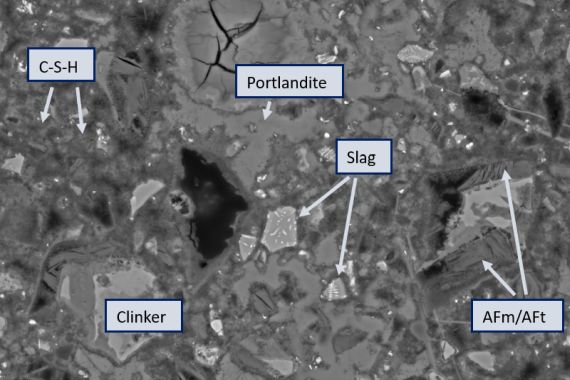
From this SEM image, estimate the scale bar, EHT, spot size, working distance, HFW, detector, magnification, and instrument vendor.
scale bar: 40000 nm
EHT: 15 kV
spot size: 5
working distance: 9.9 mm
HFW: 104 µm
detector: CBS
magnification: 4 K X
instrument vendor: FEI